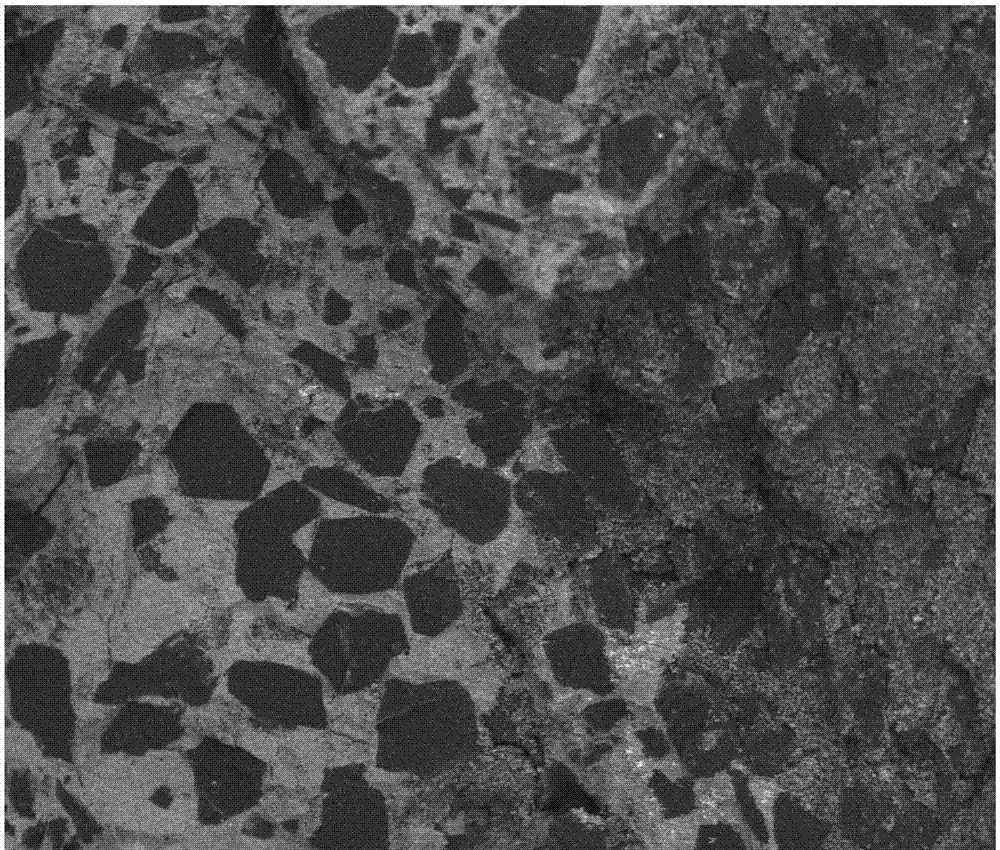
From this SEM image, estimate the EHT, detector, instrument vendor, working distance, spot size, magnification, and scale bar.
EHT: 20 kV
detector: DualBSD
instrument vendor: FEI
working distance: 14.2 mm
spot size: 3.5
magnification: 0.1 K X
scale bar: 500000 nm